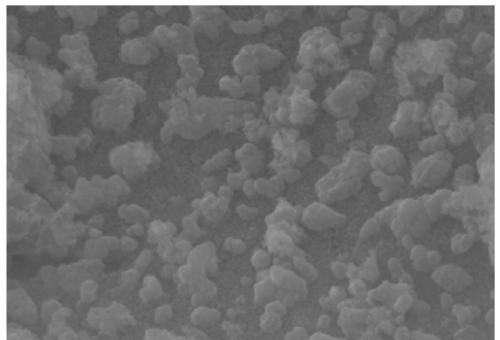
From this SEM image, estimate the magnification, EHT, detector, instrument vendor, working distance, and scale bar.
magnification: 30 K X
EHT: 15 kV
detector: InLens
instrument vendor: Zeiss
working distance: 3.3 mm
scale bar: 200 nm